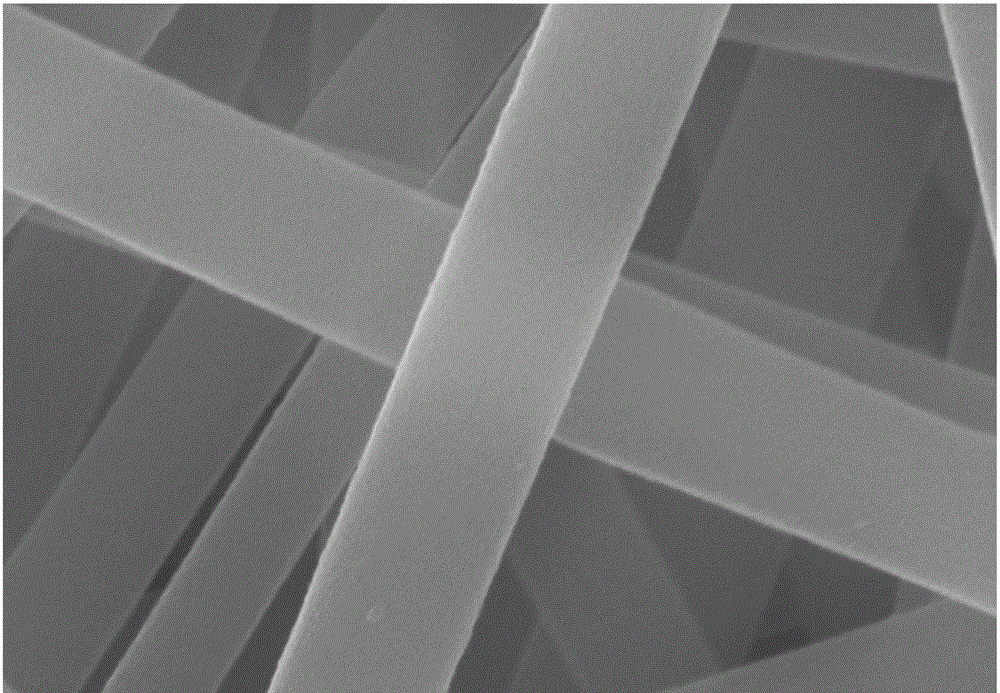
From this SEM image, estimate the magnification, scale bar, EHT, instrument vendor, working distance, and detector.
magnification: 200 K X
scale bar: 200 nm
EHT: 20 kV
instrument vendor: Zeiss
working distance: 5.9 mm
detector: InLens